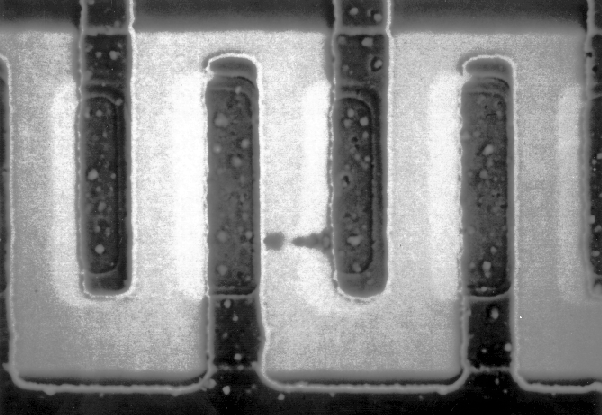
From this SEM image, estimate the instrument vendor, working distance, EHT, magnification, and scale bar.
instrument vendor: JEOL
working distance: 20 mm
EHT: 10 kV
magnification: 1.5 K X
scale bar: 10000 nm